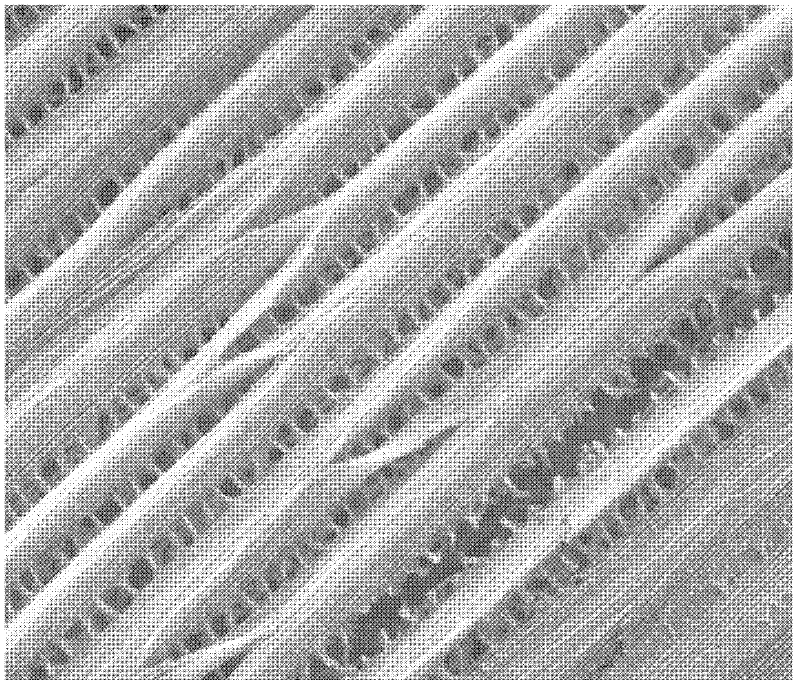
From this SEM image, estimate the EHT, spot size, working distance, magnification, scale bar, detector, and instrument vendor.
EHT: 20 kV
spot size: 2.5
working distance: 11 mm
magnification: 20 K X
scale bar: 5000 nm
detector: ETD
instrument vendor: FEI